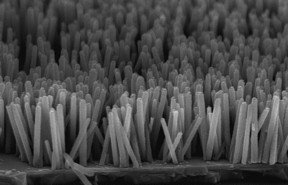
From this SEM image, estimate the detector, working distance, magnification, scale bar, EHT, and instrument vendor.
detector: SE(M,LA0)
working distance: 7.5 mm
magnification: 50 K X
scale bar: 1000 nm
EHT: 5 kV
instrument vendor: Hitachi